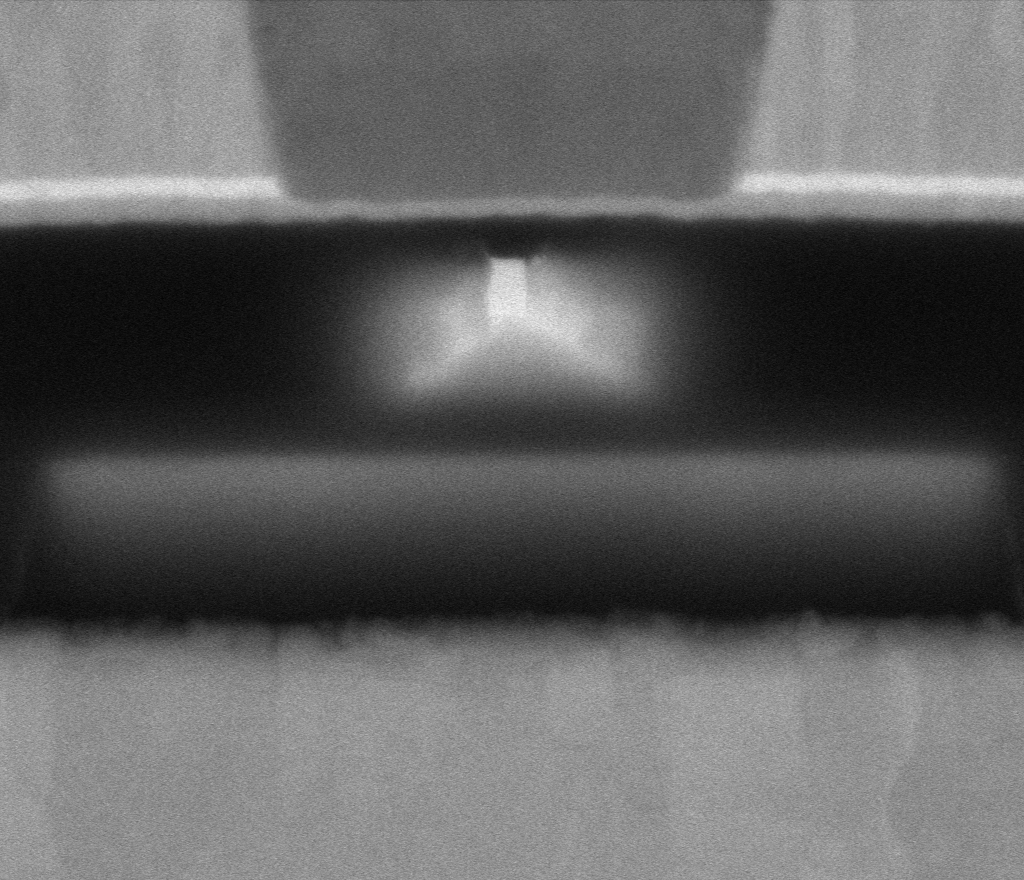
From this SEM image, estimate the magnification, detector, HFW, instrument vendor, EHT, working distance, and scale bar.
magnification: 568.609 K X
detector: MD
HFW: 0.635 µm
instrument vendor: Thermo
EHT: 5 kV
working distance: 2 mm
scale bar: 100 nm